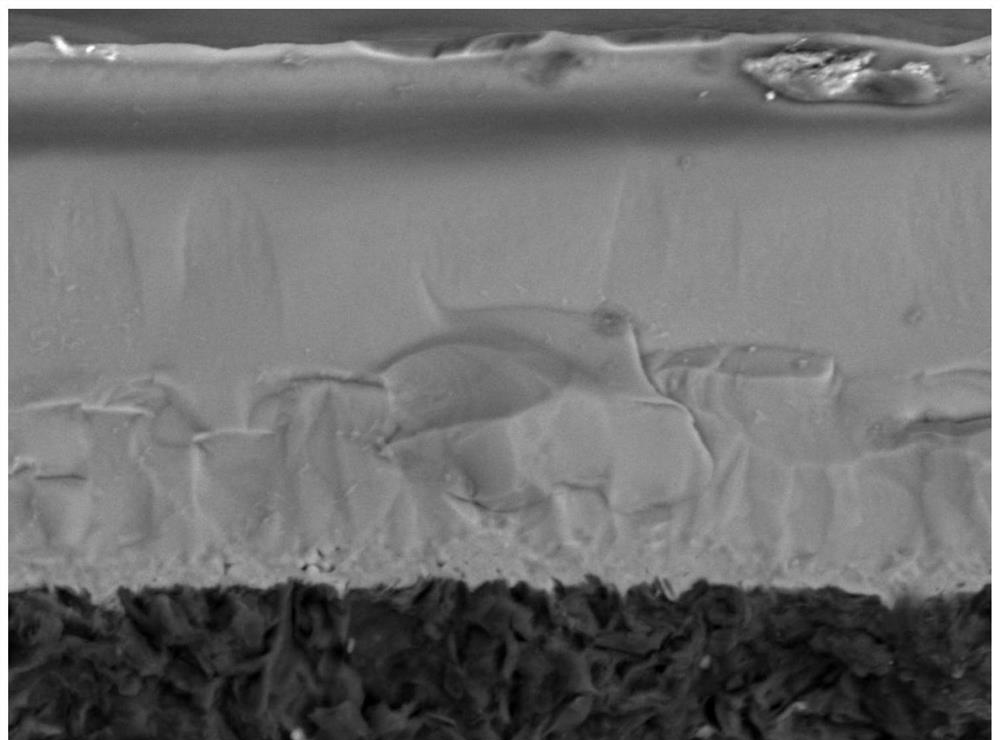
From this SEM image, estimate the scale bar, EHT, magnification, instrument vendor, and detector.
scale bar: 20000 nm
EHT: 20 kV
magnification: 0.75 K X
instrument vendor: JEOL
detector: BED-S